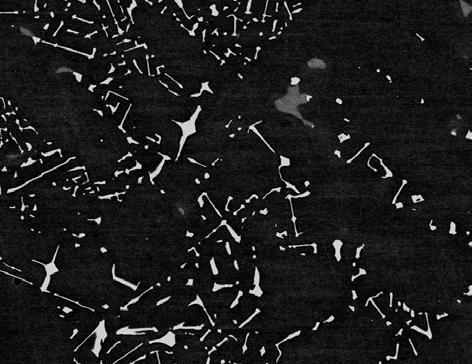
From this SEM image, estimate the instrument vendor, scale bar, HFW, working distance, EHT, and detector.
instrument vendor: FEI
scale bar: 100000 nm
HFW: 298 µm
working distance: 10.1 mm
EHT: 20 kV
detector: BSED